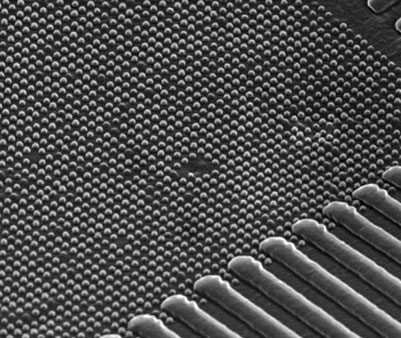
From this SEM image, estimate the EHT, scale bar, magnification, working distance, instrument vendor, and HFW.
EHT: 19 kV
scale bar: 500 nm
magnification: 150 K X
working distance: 5.2 mm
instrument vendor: FEI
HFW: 1.71 µm